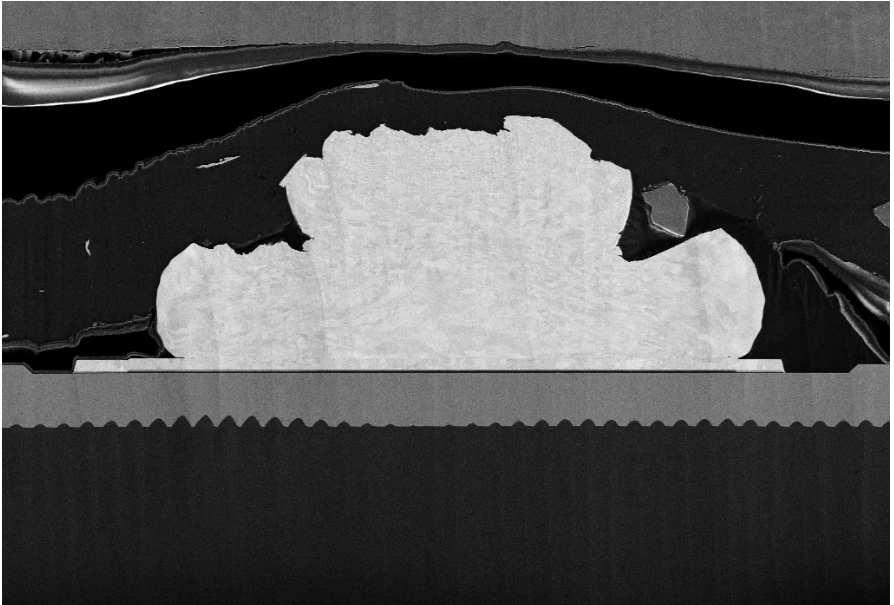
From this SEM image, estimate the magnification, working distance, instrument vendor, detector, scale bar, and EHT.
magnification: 1 K X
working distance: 4.9 mm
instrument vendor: Zeiss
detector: SE2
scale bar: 10000 nm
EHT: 5 kV